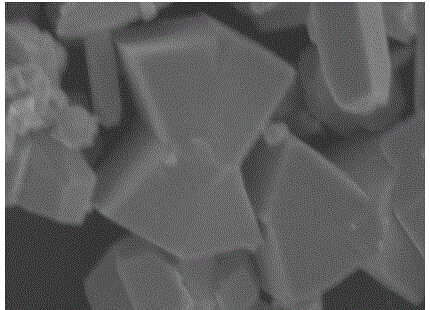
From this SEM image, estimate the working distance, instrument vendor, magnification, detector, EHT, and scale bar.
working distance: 14.6 mm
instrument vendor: Hitachi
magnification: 5 K X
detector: SE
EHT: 15 kV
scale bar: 10000 nm